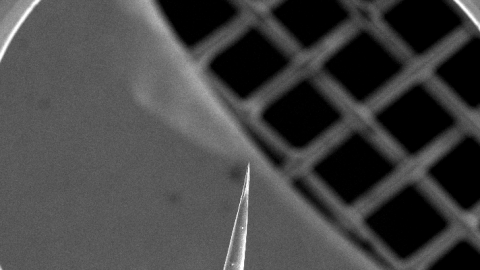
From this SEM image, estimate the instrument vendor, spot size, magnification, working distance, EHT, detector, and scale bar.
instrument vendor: FEI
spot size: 3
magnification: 0.034 K X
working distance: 7.1 mm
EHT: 15 kV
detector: SE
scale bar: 1000000 nm